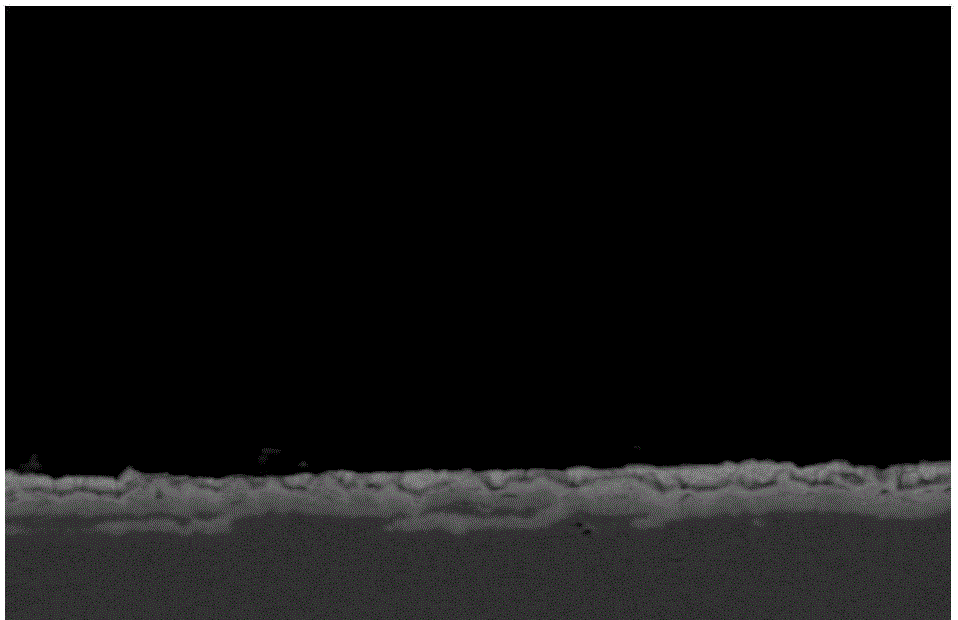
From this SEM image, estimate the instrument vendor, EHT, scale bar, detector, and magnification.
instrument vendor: JEOL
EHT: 20 kV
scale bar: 10000 nm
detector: BES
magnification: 1.3 K X